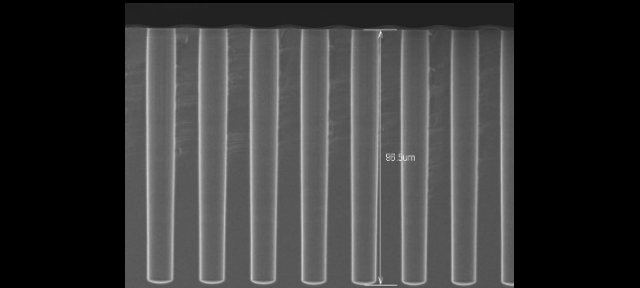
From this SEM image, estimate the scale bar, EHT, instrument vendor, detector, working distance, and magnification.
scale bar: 50000 nm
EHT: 10 kV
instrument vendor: Hitachi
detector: SE(U)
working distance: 9.2 mm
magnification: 0.8 K X